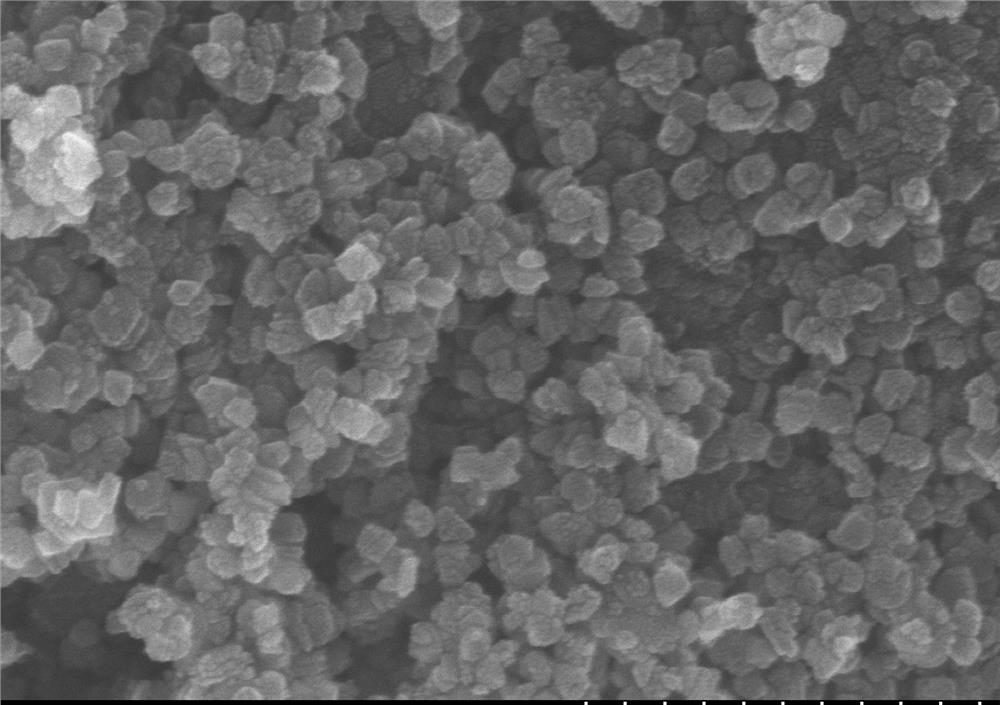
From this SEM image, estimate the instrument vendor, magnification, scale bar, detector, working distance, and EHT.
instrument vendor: Hitachi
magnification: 100 K X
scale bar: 500 nm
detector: SE(M)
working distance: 9.1 mm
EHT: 15 kV